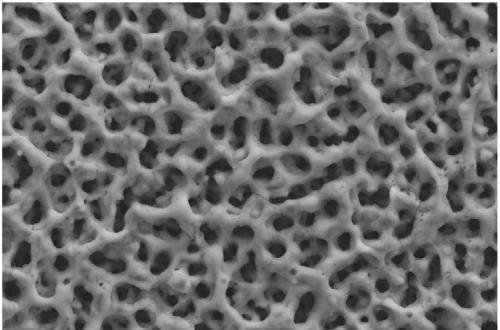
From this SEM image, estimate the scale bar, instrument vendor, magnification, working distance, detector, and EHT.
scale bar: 10000 nm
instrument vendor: Zeiss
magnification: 3 K X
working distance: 8 mm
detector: SE2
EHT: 20 kV